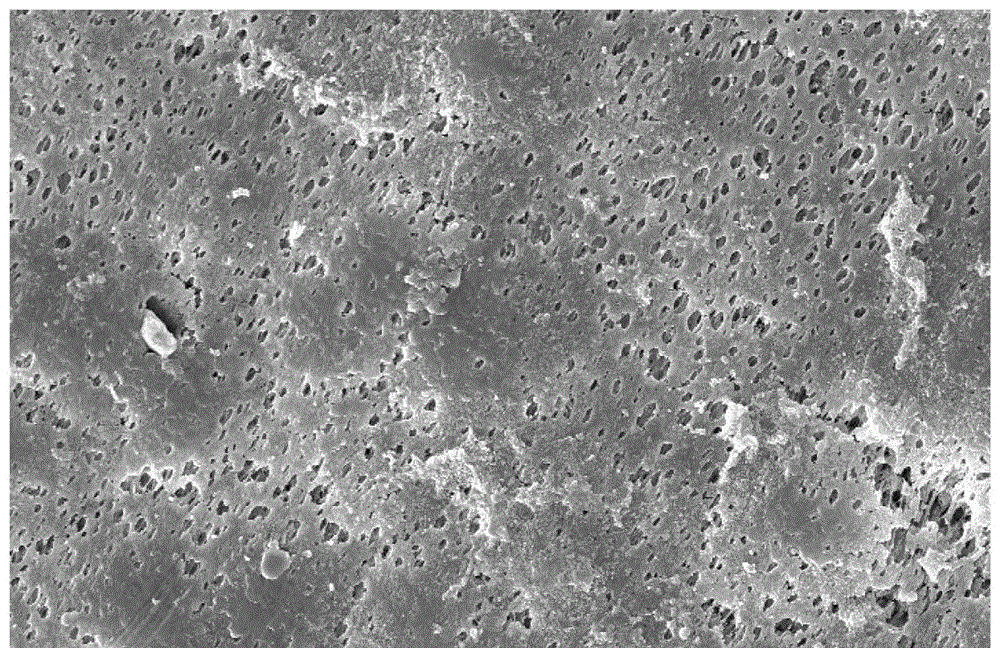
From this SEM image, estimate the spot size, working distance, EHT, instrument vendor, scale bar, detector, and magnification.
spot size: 3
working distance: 6.2 mm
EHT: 10 kV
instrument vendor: FEI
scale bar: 10000 nm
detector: SE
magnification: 2 K X